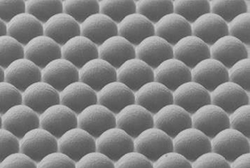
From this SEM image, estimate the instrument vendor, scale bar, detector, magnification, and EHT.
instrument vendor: JEOL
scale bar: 200000 nm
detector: BEI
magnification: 0.065 K X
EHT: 15 kV